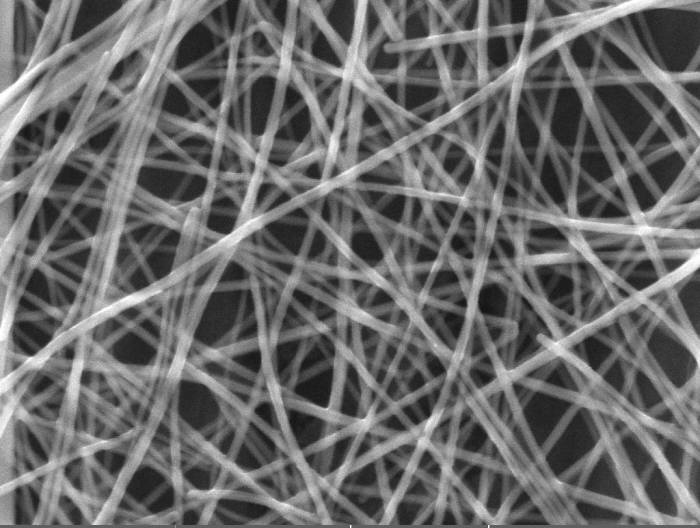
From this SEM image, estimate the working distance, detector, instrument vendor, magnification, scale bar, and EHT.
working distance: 4.96 mm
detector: InBeam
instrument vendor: Tescan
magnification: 100 K X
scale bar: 500 nm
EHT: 15 kV